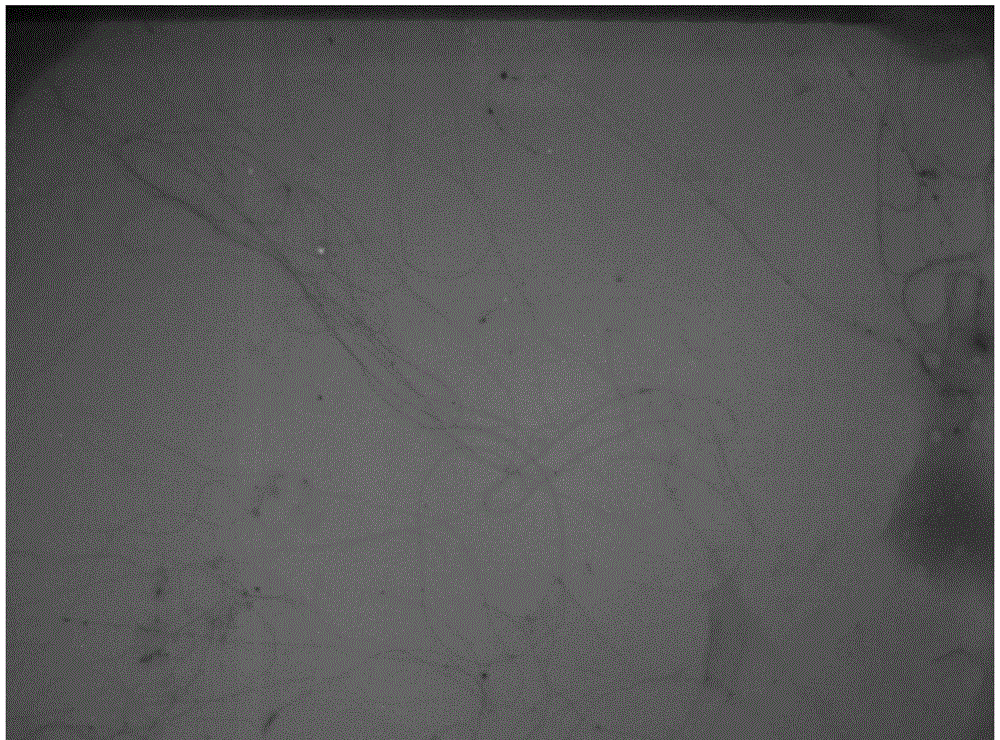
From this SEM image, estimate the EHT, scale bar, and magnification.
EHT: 100 kV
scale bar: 10010 nm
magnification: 2.5 K X